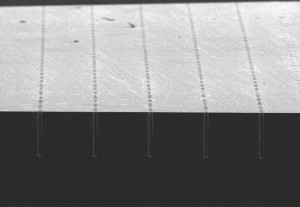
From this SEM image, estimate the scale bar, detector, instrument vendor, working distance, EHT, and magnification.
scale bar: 300000 nm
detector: SE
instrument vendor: Hitachi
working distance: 19.5 mm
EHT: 10 kV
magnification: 0.15 K X